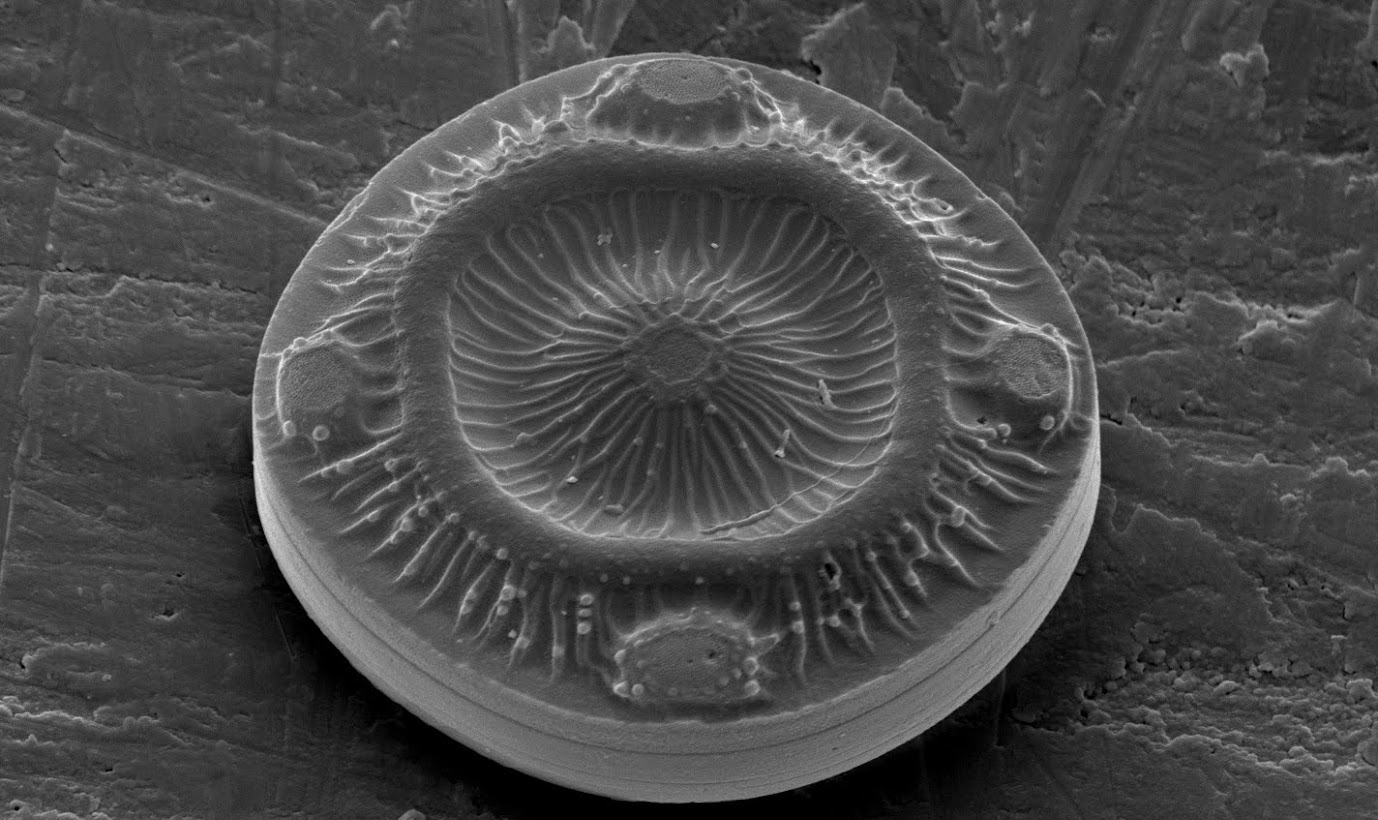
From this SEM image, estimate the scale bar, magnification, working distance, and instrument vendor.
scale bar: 10000 nm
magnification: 4 K X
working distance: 10.9 mm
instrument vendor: FEI